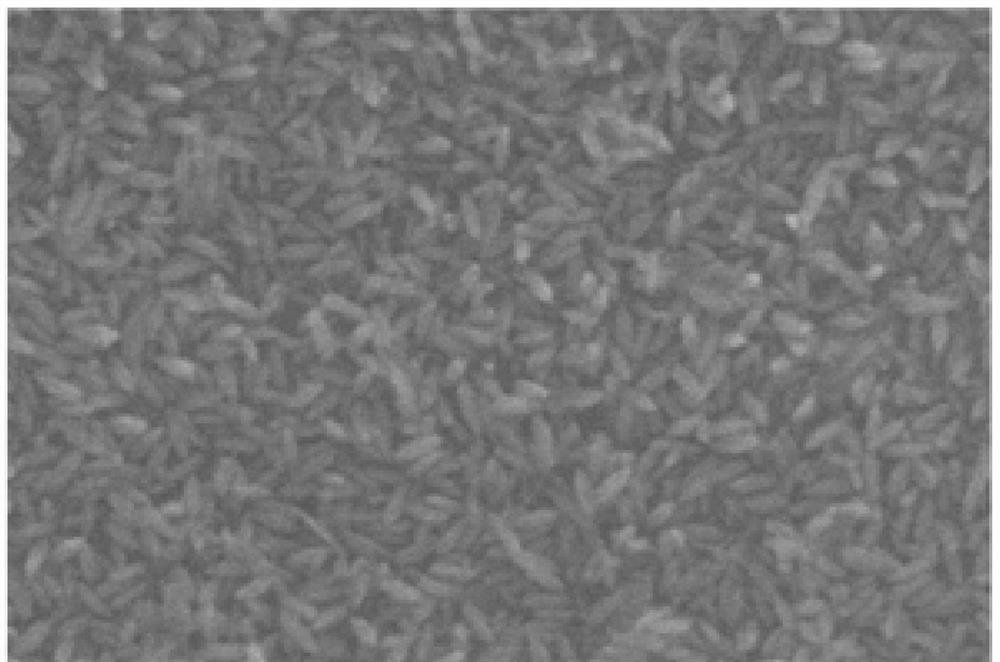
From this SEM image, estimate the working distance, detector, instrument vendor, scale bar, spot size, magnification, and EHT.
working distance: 10.1 mm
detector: SE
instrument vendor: FEI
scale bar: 500 nm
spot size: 3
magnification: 200 K X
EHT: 20 kV